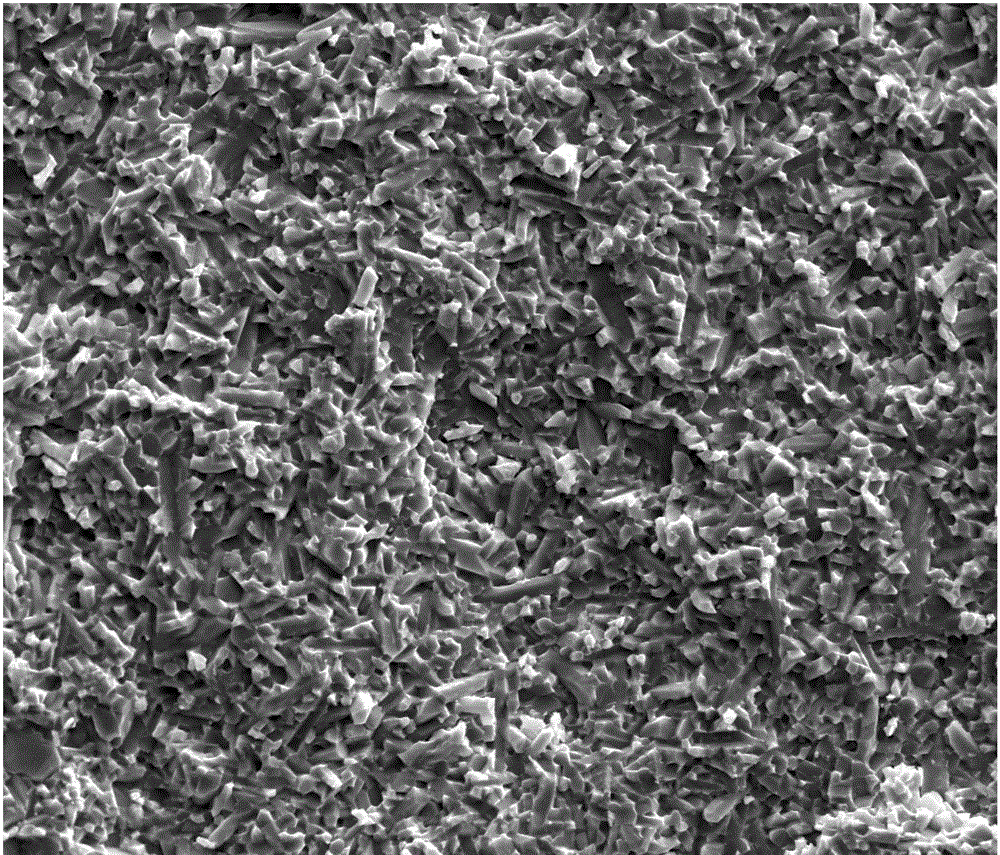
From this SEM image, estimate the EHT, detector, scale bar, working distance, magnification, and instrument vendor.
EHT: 10 kV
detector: ETD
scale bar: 20000 nm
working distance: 10.6 mm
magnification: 5 K X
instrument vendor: FEI